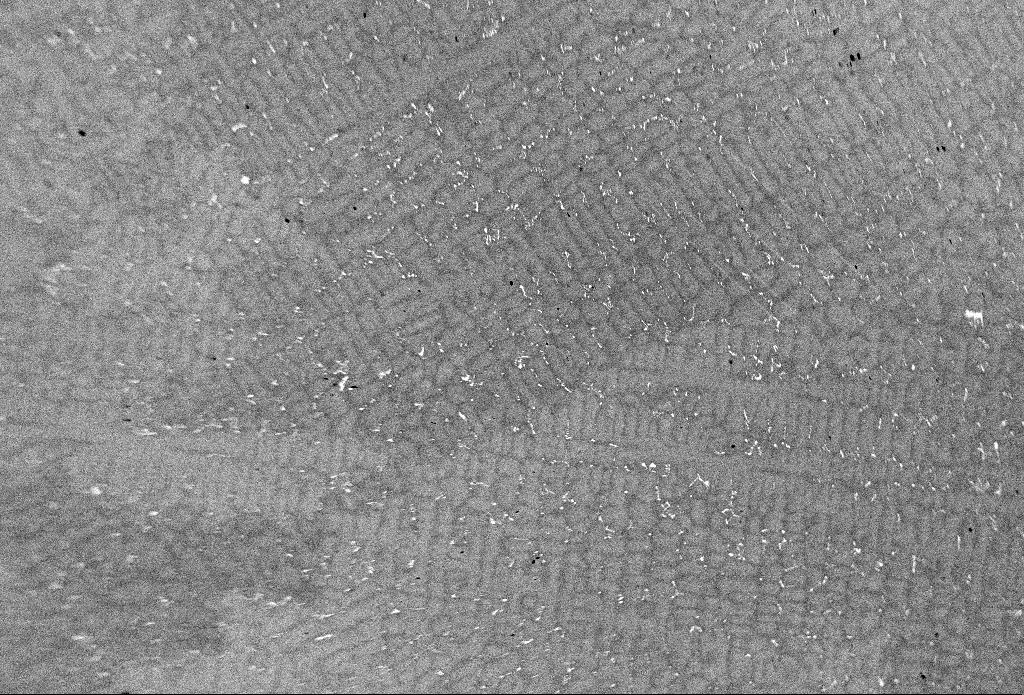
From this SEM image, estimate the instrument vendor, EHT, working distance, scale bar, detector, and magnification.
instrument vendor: Zeiss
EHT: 20 kV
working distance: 15 mm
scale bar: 100000 nm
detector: QBSD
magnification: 0.1 K X